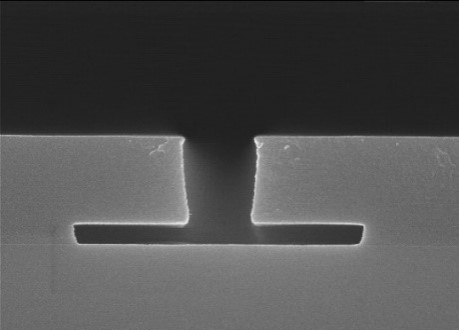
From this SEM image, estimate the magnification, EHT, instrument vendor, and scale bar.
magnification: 30 K X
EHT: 15 kV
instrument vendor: Hitachi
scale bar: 1000 nm